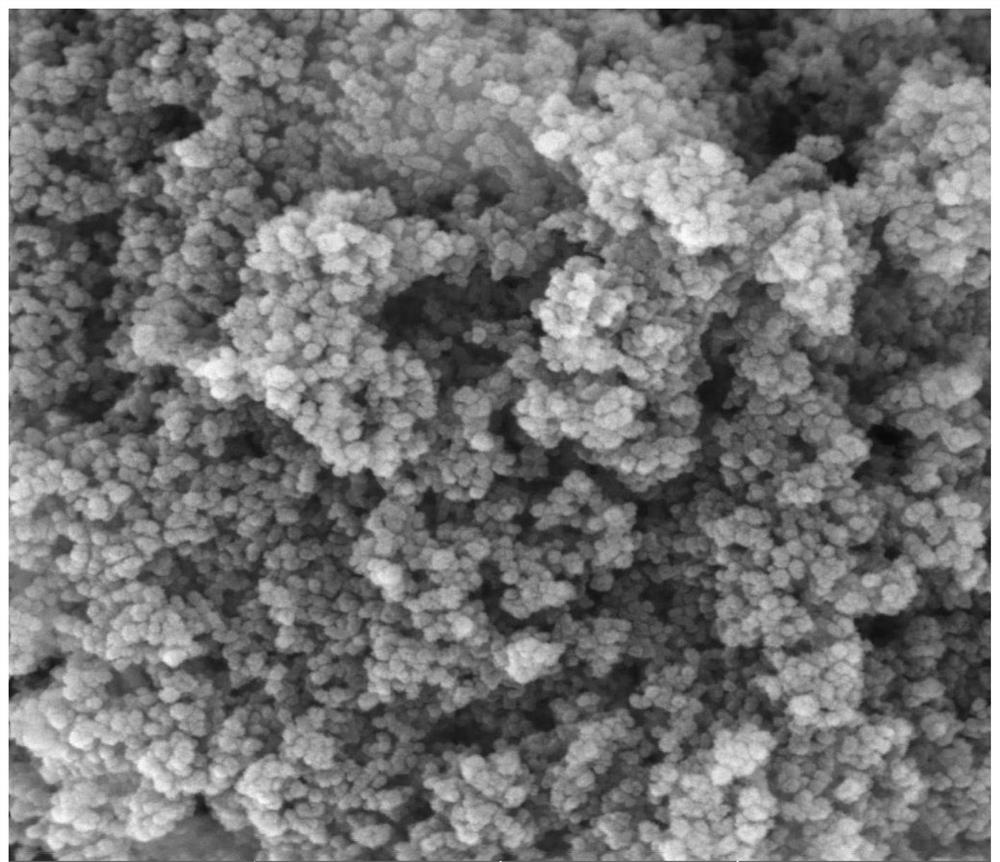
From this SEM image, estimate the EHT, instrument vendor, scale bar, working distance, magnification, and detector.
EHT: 15 kV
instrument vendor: Tescan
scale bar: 500 nm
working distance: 5.01 mm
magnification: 100 K X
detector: In-Beam SE